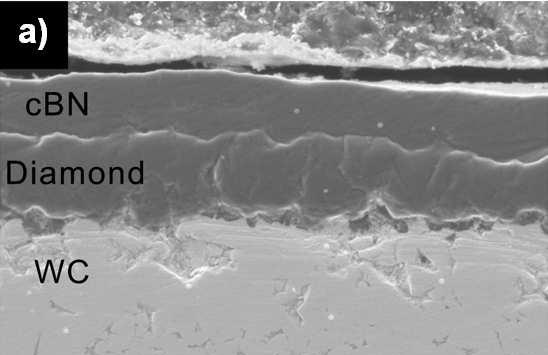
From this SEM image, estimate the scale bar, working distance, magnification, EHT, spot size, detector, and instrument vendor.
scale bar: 5000 nm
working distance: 10.1 mm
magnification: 5 K X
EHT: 5 kV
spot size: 3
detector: SE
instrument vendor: FEI